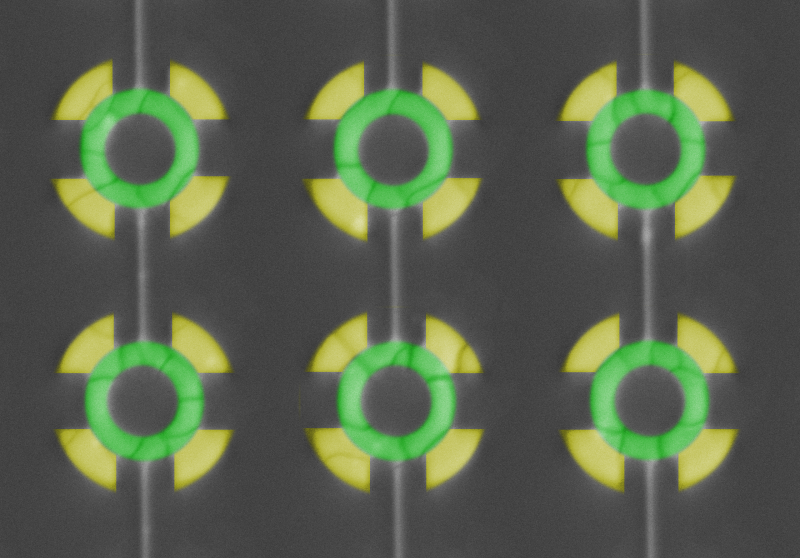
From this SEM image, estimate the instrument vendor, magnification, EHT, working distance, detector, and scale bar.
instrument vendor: Hitachi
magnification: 2 K X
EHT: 15 kV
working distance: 9 mm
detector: BSE M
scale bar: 20000 nm